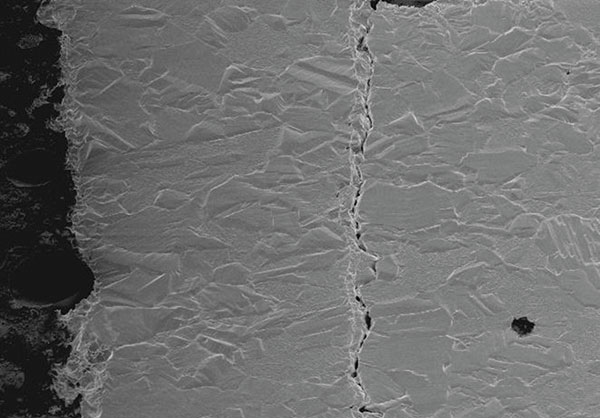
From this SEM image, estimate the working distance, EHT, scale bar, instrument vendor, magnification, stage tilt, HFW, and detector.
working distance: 4 mm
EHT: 2 kV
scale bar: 10000 nm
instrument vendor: FEI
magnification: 5 K X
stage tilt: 52°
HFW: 41.4 µm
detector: SE